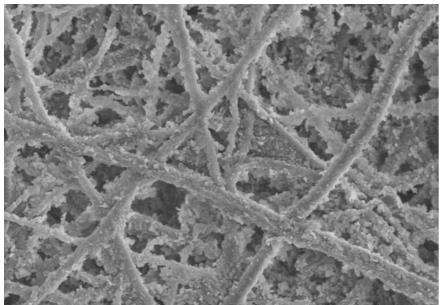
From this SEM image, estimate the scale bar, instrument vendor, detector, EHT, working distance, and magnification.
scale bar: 10000 nm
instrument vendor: Hitachi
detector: SE(M)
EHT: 5 kV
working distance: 11.3 mm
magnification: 5 K X